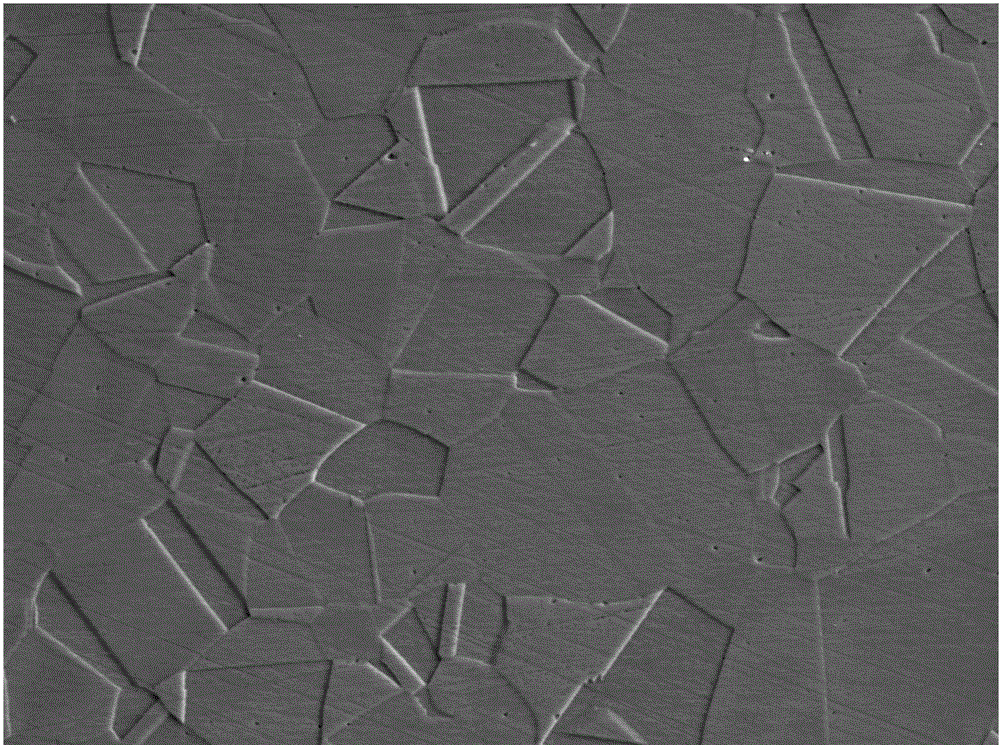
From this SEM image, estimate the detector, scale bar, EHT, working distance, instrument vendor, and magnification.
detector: SEI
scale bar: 10000 nm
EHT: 20 kV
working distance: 10.8 mm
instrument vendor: JEOL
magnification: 1 K X